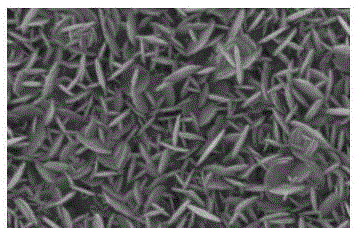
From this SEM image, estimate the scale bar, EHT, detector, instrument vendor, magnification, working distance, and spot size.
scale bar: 500 nm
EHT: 12 kV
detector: SE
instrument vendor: FEI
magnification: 50 K X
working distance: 4.4 mm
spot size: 3